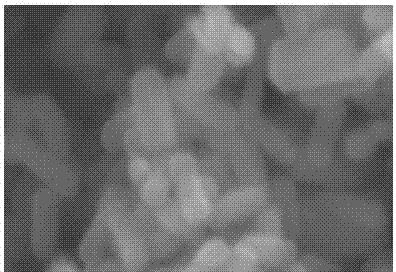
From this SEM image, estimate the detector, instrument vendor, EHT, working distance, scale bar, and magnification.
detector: SE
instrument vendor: Hitachi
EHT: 20 kV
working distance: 8.2 mm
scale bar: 1000 nm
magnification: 42 K X